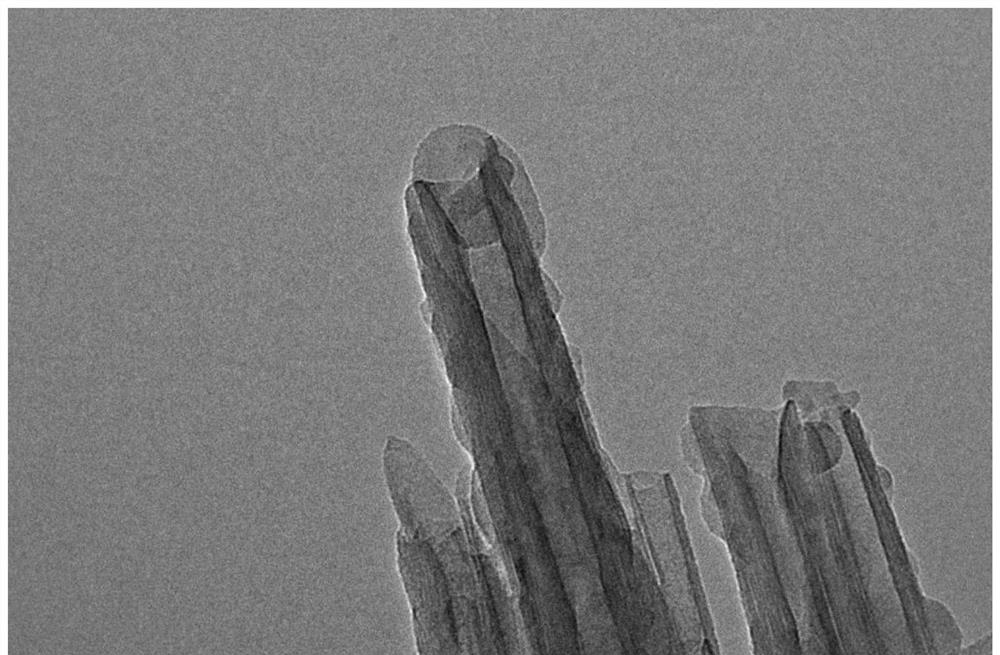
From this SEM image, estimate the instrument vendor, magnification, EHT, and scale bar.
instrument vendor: JEOL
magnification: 100 K X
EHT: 200 kV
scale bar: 100 nm